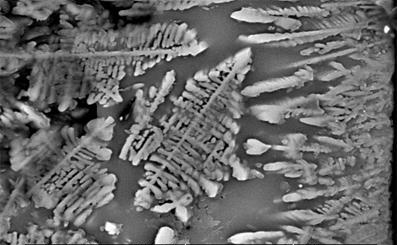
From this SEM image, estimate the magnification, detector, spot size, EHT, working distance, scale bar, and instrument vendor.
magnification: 2.5 K X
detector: BSE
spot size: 5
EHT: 20 kV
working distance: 10.2 mm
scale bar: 10000 nm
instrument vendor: FEI